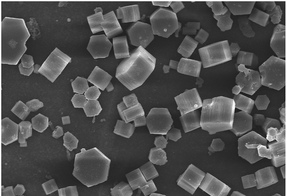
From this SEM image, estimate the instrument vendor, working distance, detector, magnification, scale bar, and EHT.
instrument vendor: Hitachi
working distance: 9.5 mm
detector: SE(M)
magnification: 3 K X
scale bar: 10000 nm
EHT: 10 kV